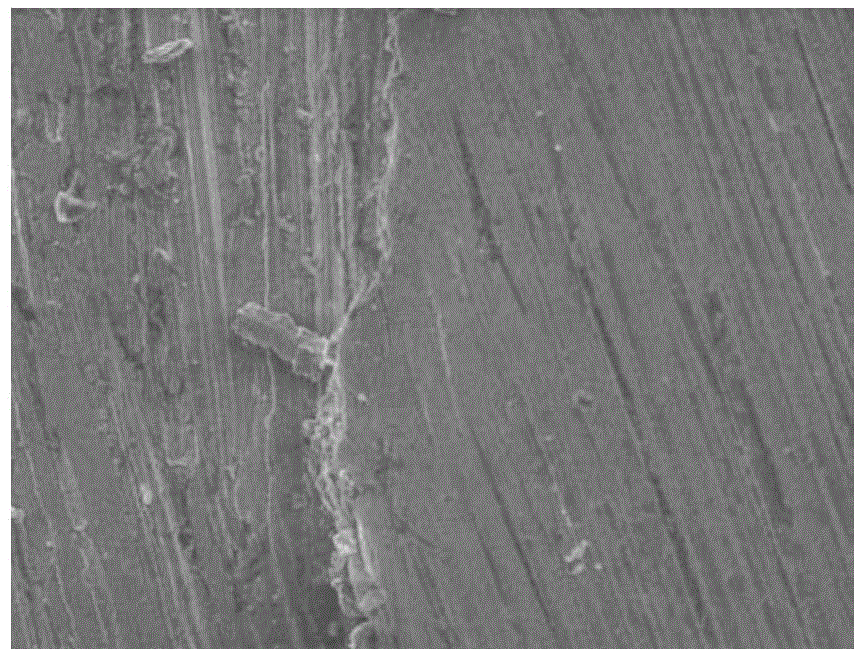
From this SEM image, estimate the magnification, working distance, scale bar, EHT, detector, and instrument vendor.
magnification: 0.3 K X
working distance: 10.6 mm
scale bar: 200000 nm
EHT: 20 kV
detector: ETD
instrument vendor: FEI